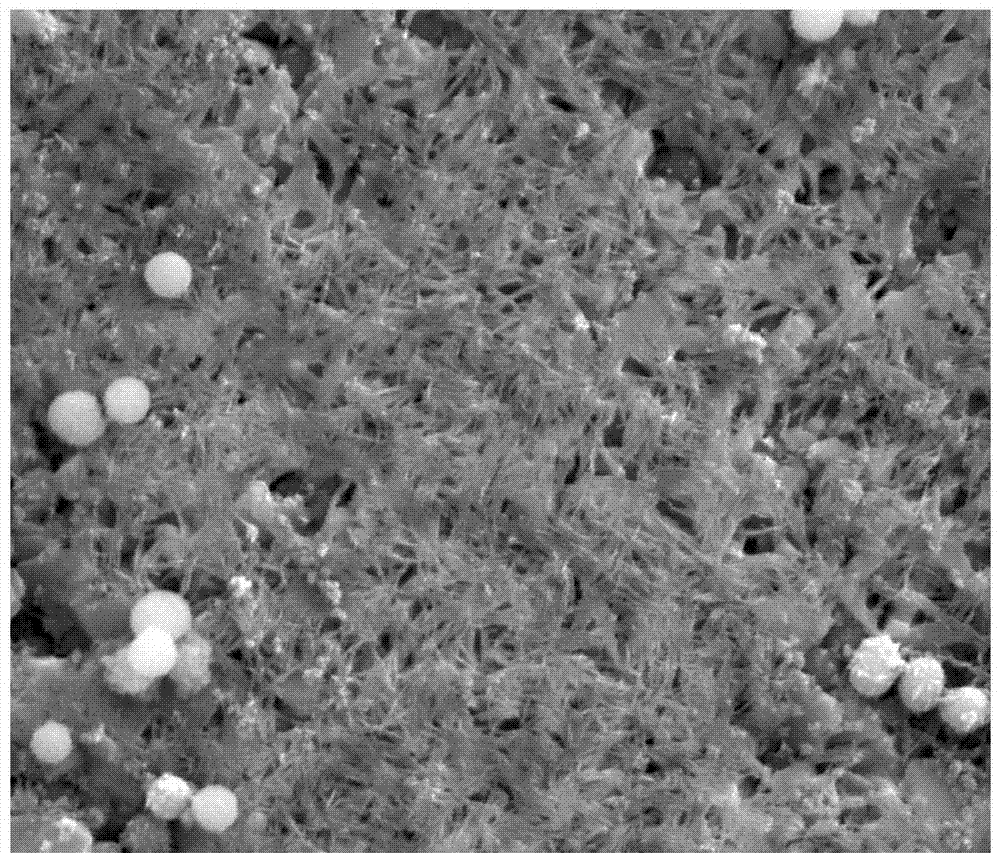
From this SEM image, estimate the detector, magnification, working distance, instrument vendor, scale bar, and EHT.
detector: ETD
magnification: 30 K X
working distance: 6.3 mm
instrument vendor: FEI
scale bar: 3000 nm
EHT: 10 kV